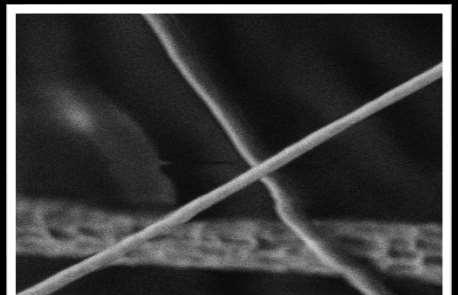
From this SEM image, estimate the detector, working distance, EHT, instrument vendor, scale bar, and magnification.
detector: SE2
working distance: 3.4 mm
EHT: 1.48 kV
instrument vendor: Zeiss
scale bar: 200 nm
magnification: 75.52 K X